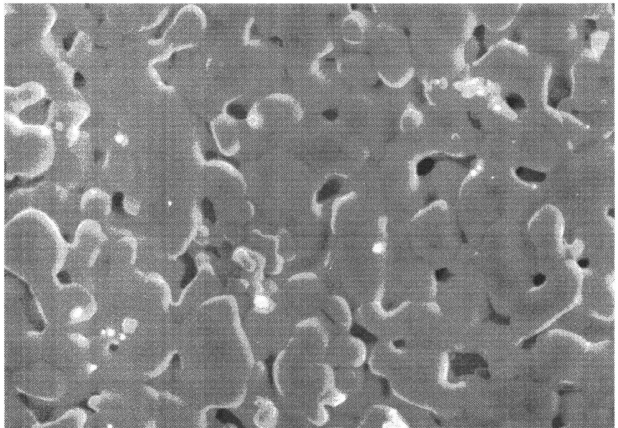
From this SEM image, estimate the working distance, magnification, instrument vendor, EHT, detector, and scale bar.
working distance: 10.4 mm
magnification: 10 K X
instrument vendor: Hitachi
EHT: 15 kV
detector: SE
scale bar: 5000 nm